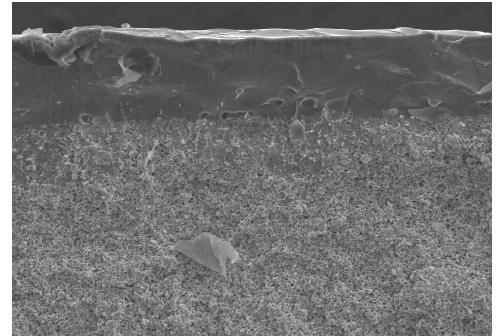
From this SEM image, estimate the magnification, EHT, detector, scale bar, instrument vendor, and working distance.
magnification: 0.5 K X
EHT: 15 kV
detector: SE(U)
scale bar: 100000 nm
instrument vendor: Hitachi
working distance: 12 mm